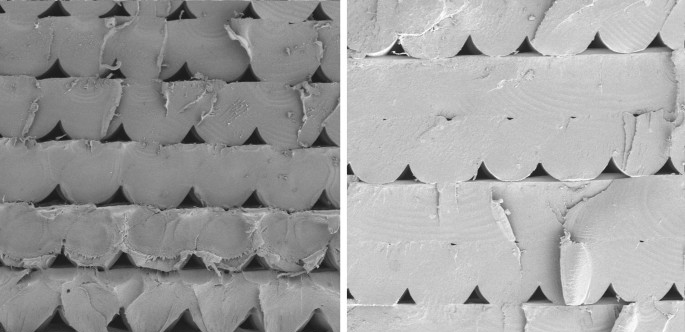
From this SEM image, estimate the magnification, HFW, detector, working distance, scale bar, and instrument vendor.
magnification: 0.1 K X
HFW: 2080 µm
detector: SE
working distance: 18.16 mm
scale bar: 500000 nm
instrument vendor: Tescan